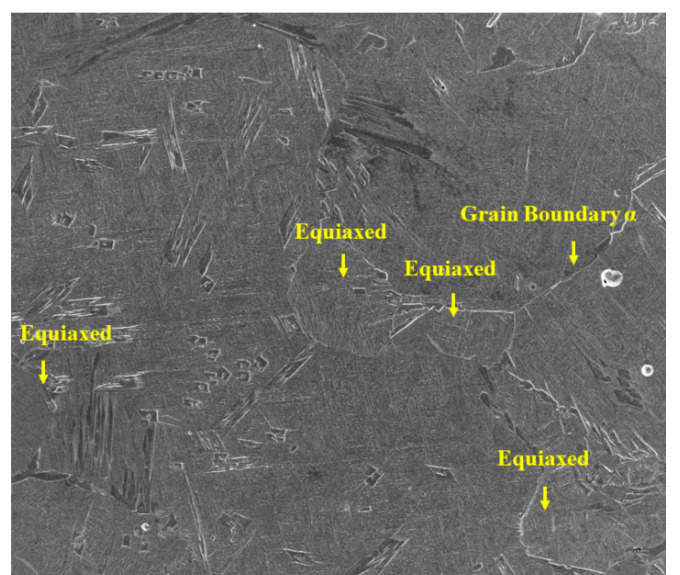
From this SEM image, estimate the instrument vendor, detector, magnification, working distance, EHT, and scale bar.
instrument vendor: FEI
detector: ETD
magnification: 0.12 K X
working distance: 9.6 mm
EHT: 20 kV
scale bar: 500000 nm